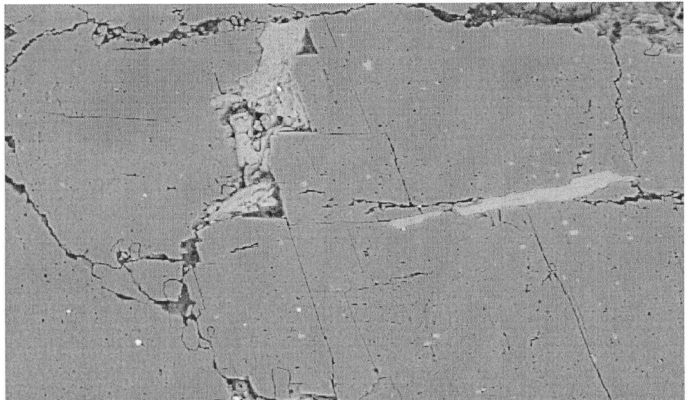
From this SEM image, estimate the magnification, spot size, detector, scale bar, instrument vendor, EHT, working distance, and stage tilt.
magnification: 0.2 K X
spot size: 5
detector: BSE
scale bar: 100000 nm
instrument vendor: FEI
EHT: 25 kV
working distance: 10.1 mm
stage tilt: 0°